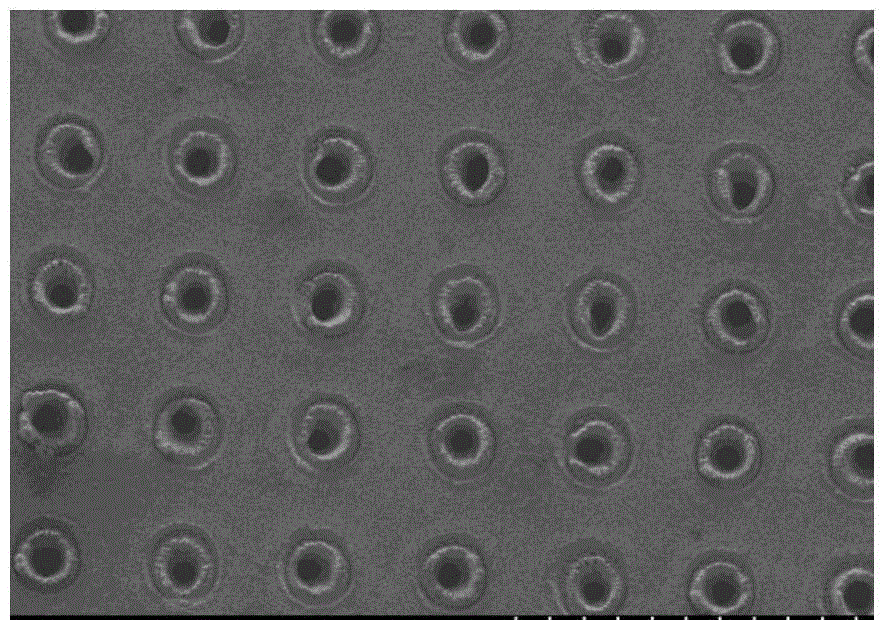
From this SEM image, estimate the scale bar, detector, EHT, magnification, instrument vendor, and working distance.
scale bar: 500000 nm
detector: SE(M)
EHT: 5 kV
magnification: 0.1 K X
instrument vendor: Hitachi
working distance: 8.7 mm